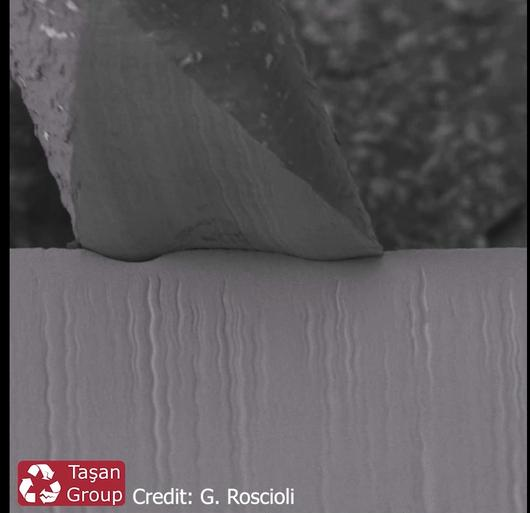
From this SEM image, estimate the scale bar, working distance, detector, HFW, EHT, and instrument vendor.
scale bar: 50000 nm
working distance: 14.89 mm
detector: SE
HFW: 198 µm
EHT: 2 kV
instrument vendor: Tescan